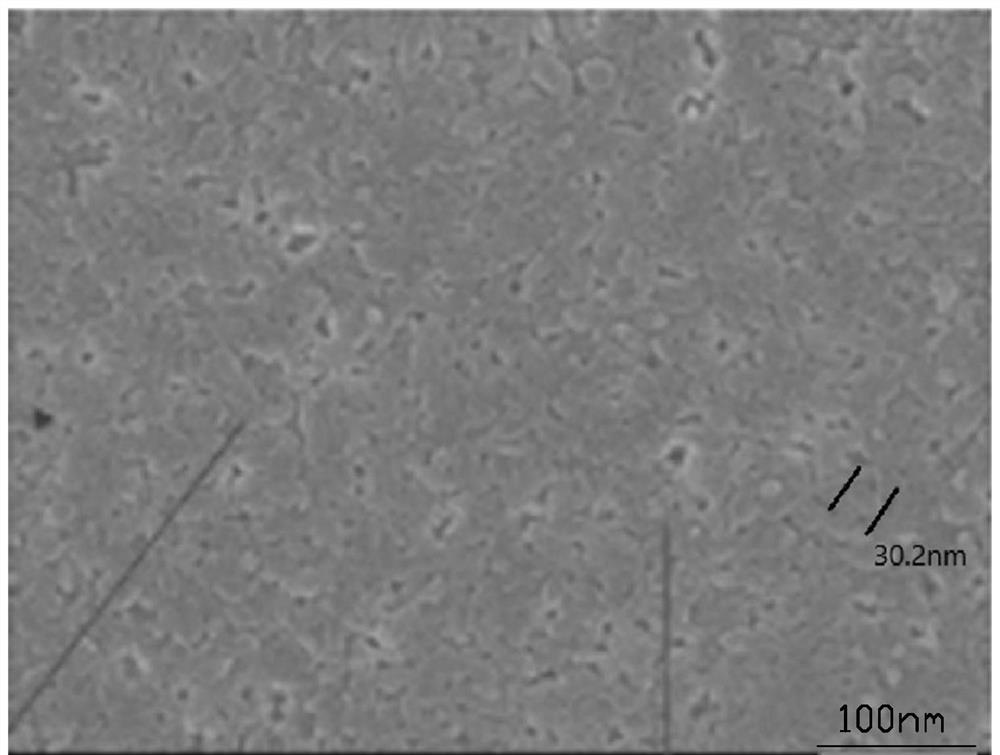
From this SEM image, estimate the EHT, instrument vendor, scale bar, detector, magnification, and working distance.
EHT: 15 kV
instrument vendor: JEOL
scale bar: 100 nm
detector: SEI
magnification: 50 K X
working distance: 11.5 mm